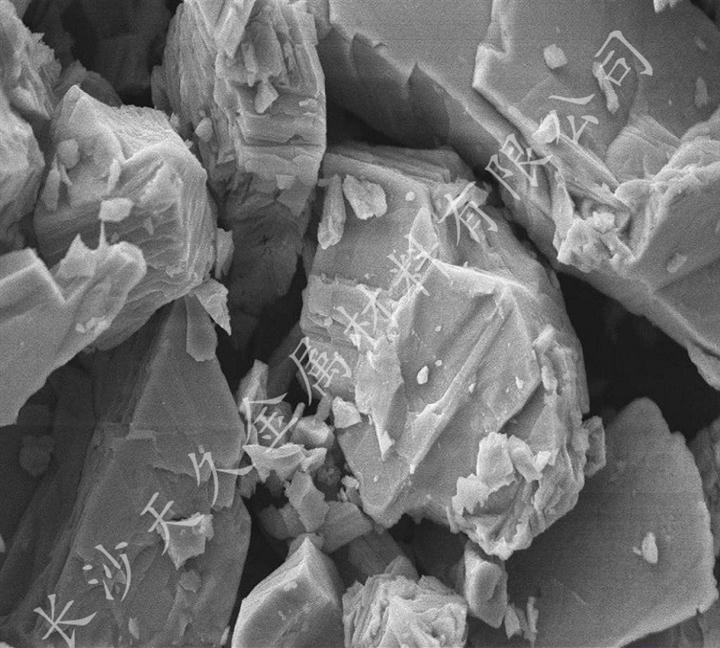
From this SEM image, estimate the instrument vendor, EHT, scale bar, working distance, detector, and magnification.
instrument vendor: Zeiss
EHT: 10 kV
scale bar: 2000 nm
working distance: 7 mm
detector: SE1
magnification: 10 K X